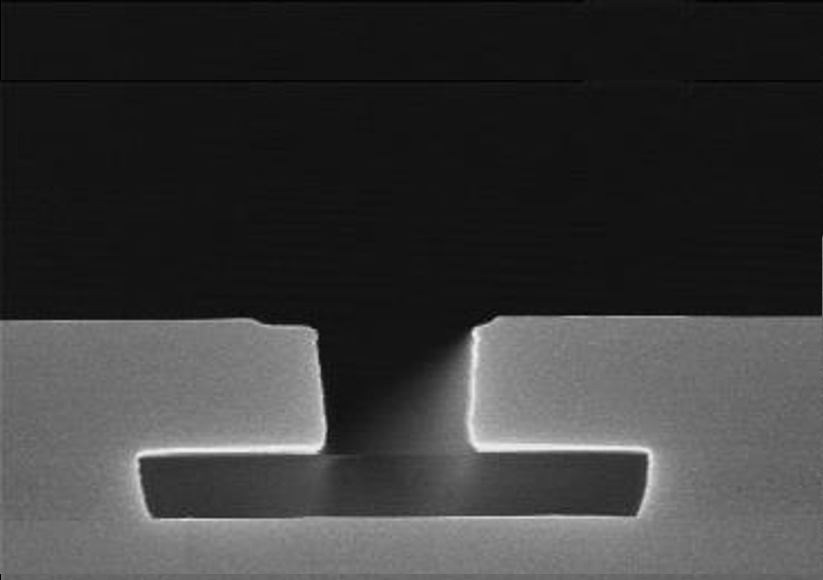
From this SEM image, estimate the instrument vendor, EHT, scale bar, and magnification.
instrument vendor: JEOL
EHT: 15 kV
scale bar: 1200 nm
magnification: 25 K X